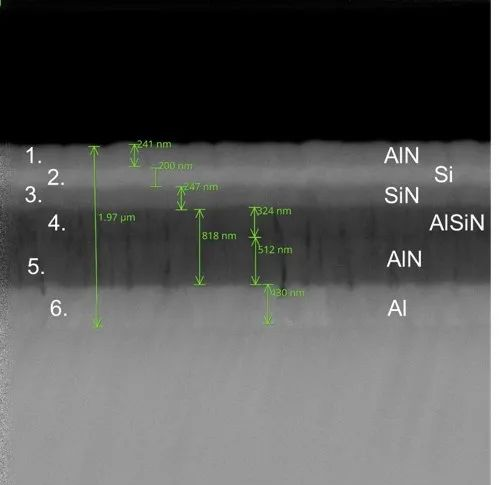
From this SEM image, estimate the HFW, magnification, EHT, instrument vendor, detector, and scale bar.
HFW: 5.37 µm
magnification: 50 K X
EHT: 10 kV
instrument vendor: Thermo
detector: BSD Full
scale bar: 1000 nm